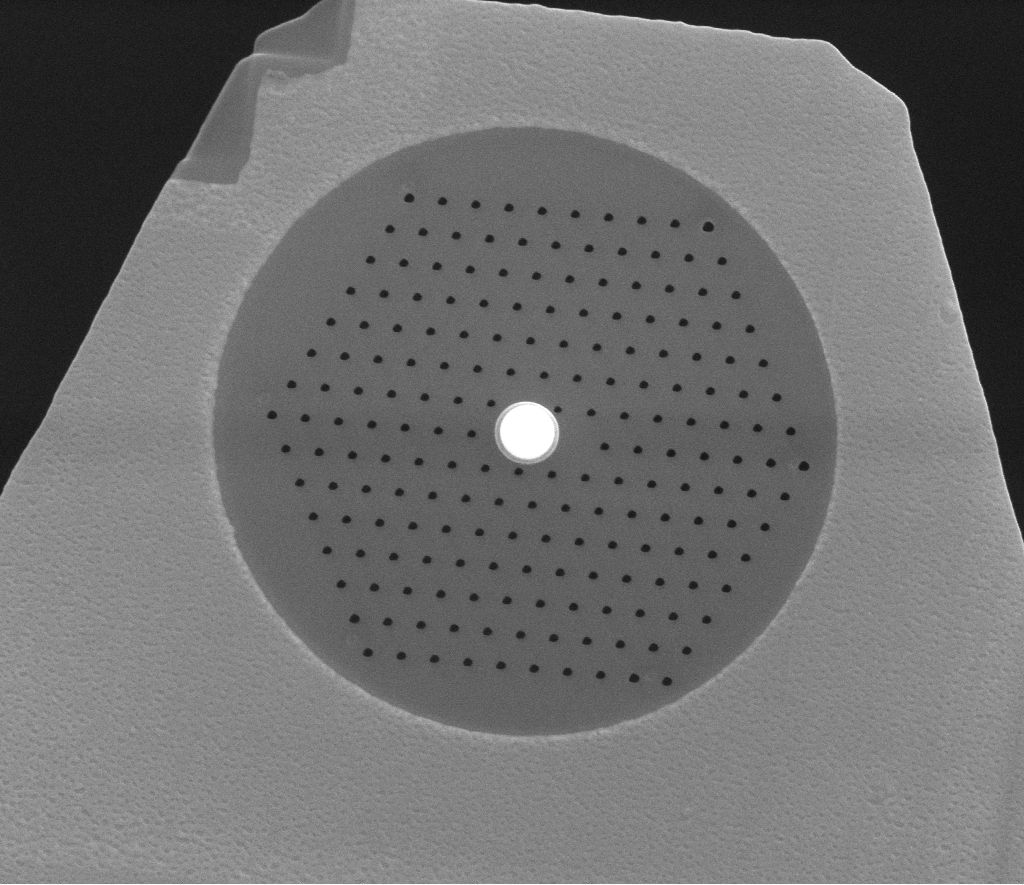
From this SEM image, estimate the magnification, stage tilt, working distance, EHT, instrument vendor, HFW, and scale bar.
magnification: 17.5 K X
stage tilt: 0°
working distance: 4.6 mm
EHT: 20 kV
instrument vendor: FEI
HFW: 8.53 µm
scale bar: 3000 nm